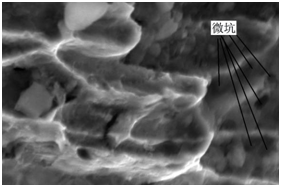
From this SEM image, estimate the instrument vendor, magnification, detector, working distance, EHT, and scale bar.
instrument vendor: Hitachi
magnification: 10 K X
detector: SE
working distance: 7.7 mm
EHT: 15 kV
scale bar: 5000 nm